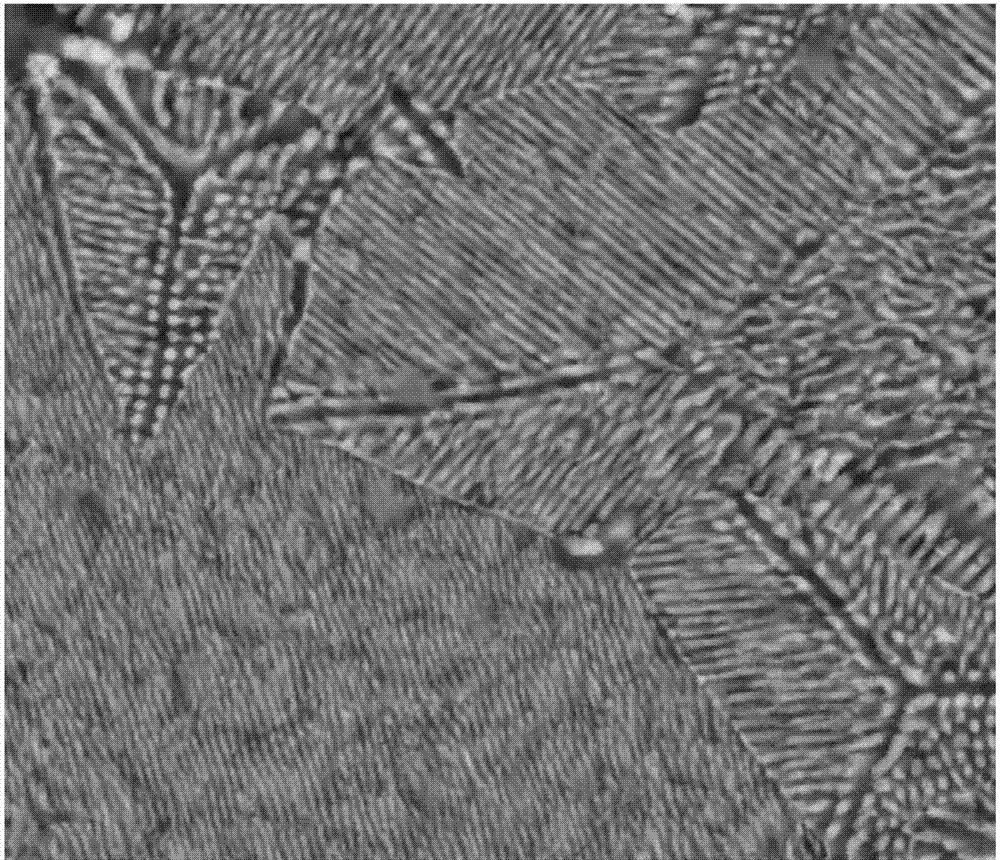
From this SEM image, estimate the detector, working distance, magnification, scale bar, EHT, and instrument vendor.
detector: Z Cont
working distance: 10.7 mm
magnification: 5 K X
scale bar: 20000 nm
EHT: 30 kV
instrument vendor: FEI